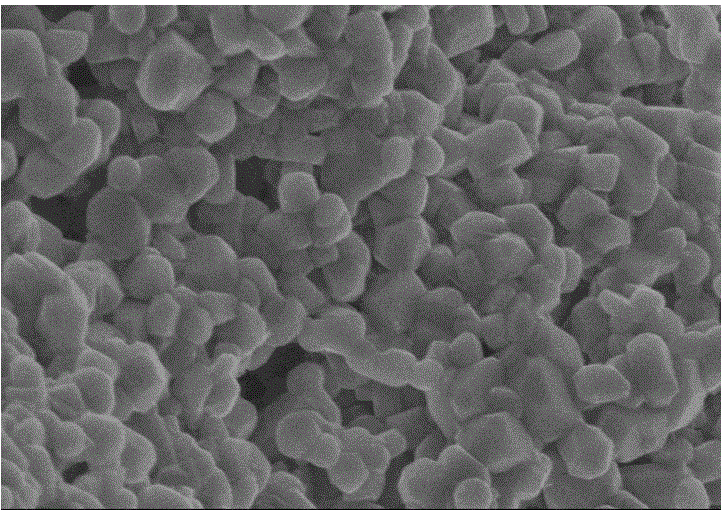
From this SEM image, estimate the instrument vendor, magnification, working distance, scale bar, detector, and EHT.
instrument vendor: Hitachi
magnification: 50 K X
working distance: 8.9 mm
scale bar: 1000 nm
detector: SE(U)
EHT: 4 kV